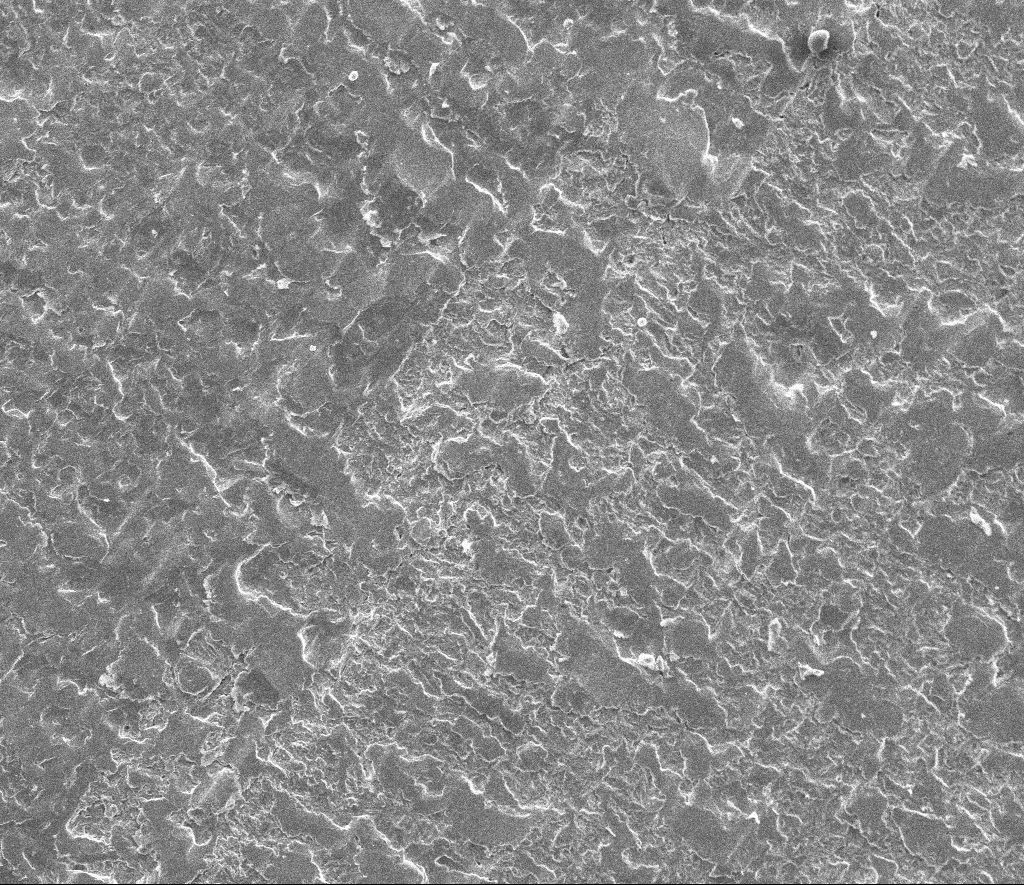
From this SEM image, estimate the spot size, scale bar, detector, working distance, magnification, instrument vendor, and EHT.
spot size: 2.5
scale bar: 200000 nm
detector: ETD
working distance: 11.6 mm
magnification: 0.2 K X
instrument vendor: FEI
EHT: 20 kV